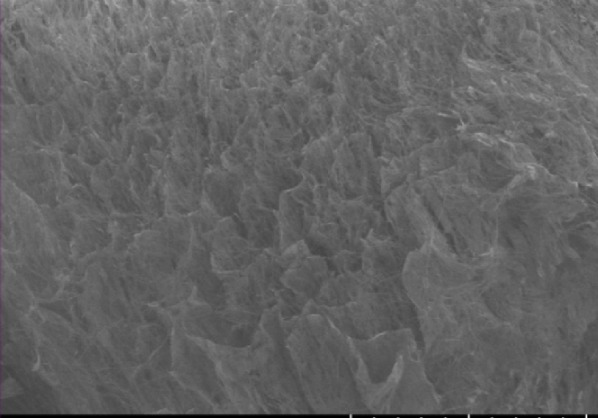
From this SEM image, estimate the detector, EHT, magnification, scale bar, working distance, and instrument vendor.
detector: LM(UL)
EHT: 1 kV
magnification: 0.1 K X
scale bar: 500000 nm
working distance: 8.6 mm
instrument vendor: Hitachi